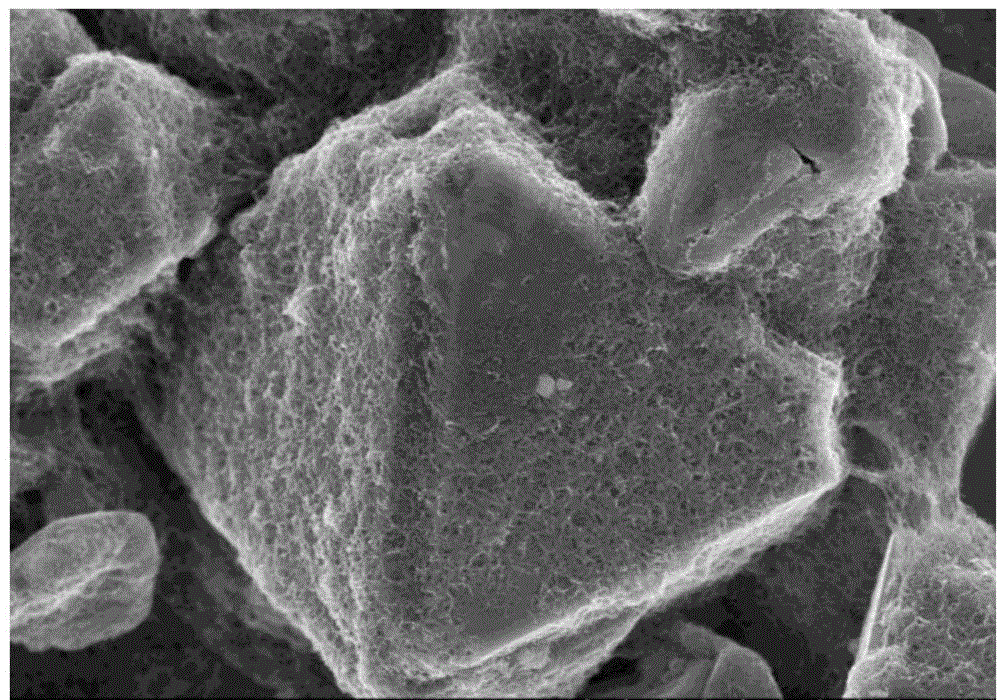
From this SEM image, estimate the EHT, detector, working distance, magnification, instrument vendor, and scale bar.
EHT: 8 kV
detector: SE(M)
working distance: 8.5 mm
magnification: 10 K X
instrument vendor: Hitachi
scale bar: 5000 nm